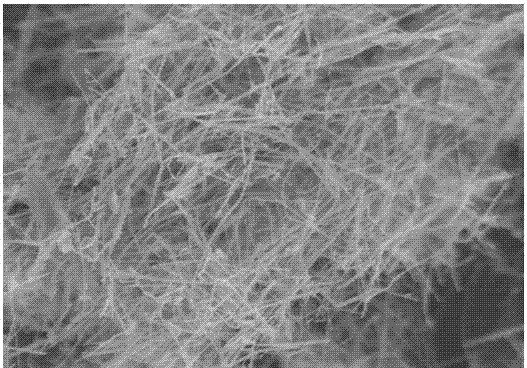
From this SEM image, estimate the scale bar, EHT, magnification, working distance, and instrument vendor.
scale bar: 10000 nm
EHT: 10 kV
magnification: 4 K X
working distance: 8.8 mm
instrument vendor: Hitachi